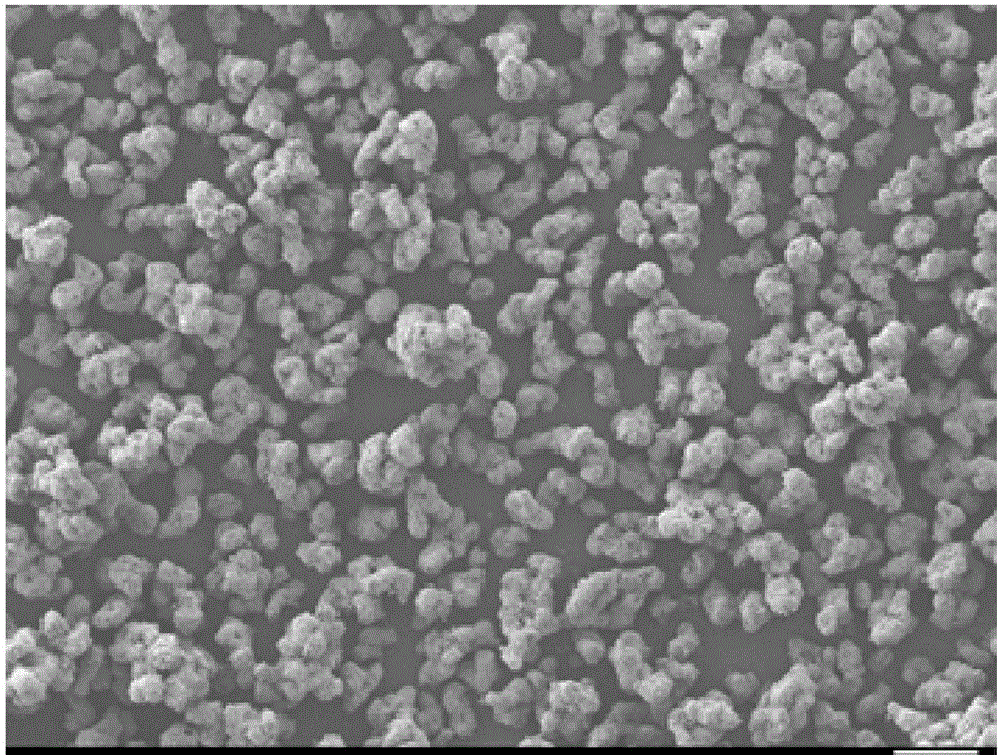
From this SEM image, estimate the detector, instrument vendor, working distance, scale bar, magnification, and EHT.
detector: LEI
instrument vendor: JEOL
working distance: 8 mm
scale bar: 100000 nm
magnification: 0.1 K X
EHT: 5 kV